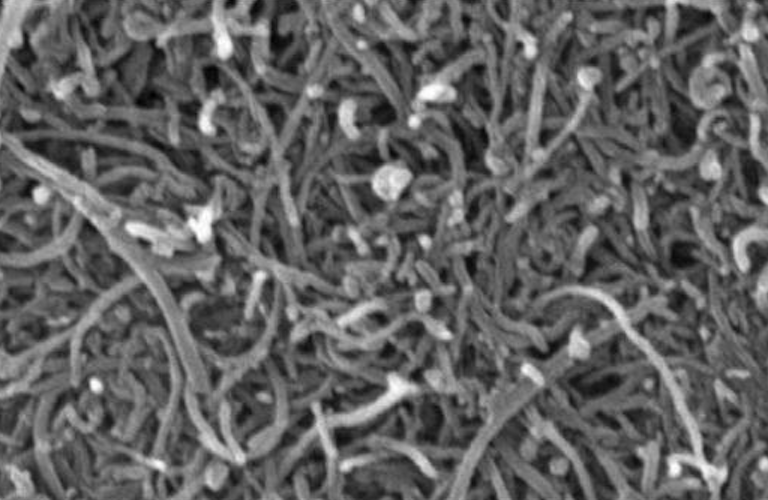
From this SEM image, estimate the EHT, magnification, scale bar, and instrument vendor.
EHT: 20 kV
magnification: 30 K X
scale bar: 500 nm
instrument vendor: JEOL